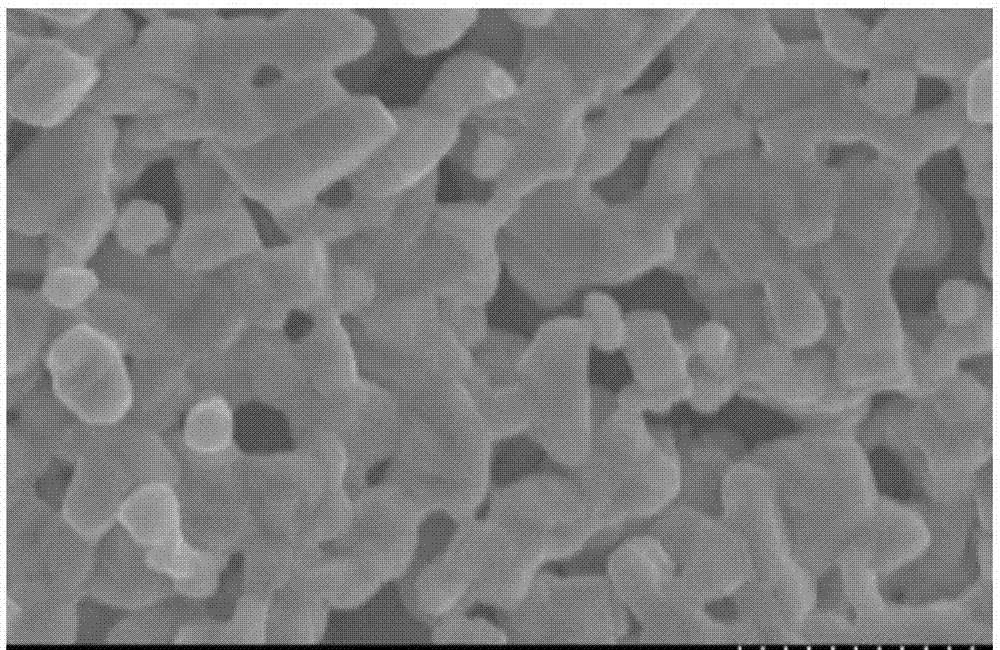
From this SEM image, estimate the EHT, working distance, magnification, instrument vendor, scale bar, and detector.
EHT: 10 kV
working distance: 9.8 mm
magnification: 30 K X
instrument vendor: Hitachi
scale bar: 1000 nm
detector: SE(M)